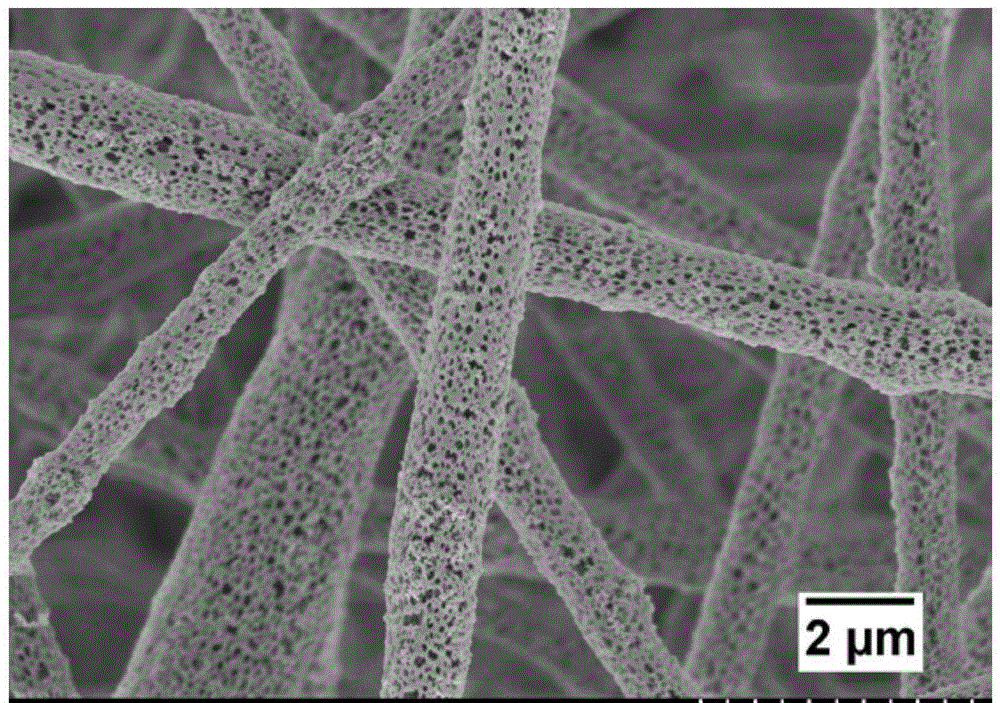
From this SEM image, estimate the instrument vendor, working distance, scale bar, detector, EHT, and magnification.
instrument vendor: Hitachi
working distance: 10.1 mm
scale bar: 5000 nm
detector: SE(M)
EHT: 3 kV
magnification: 7 K X